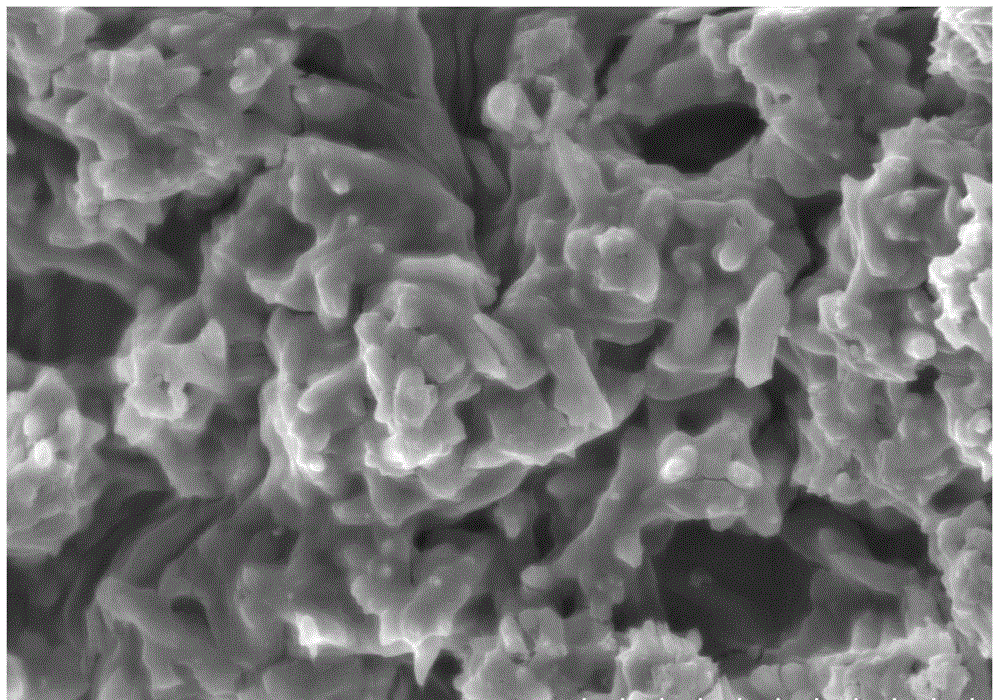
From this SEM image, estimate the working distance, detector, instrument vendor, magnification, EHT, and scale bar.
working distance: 9.1 mm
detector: SE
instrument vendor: Hitachi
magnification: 5 K X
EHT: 10 kV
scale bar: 10000 nm